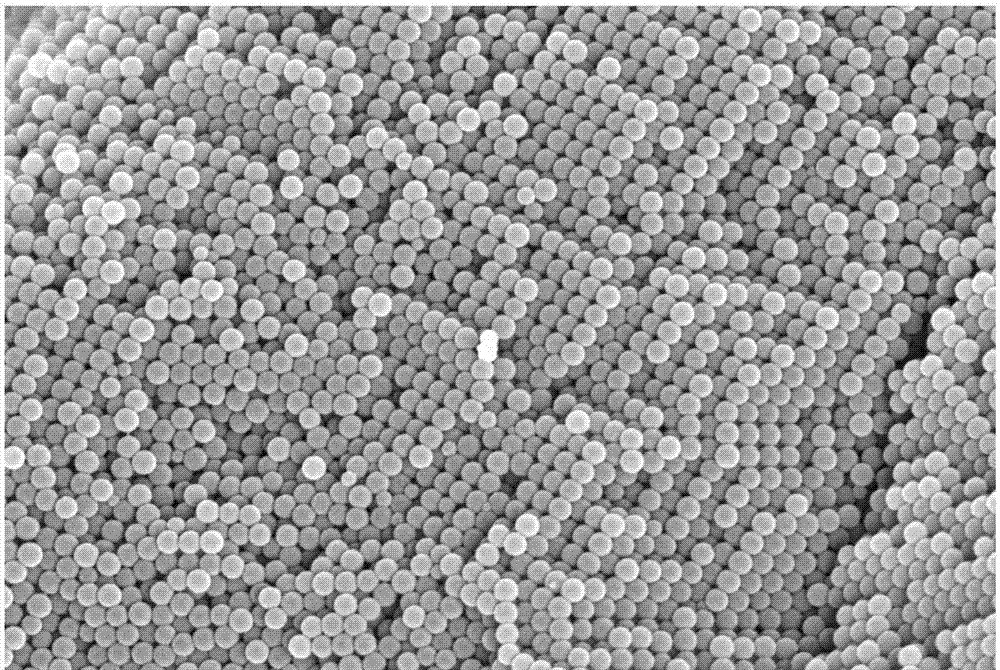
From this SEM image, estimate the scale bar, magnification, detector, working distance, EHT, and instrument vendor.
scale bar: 200 nm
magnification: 20 K X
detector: InLens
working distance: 5.5 mm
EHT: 5 kV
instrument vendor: Zeiss